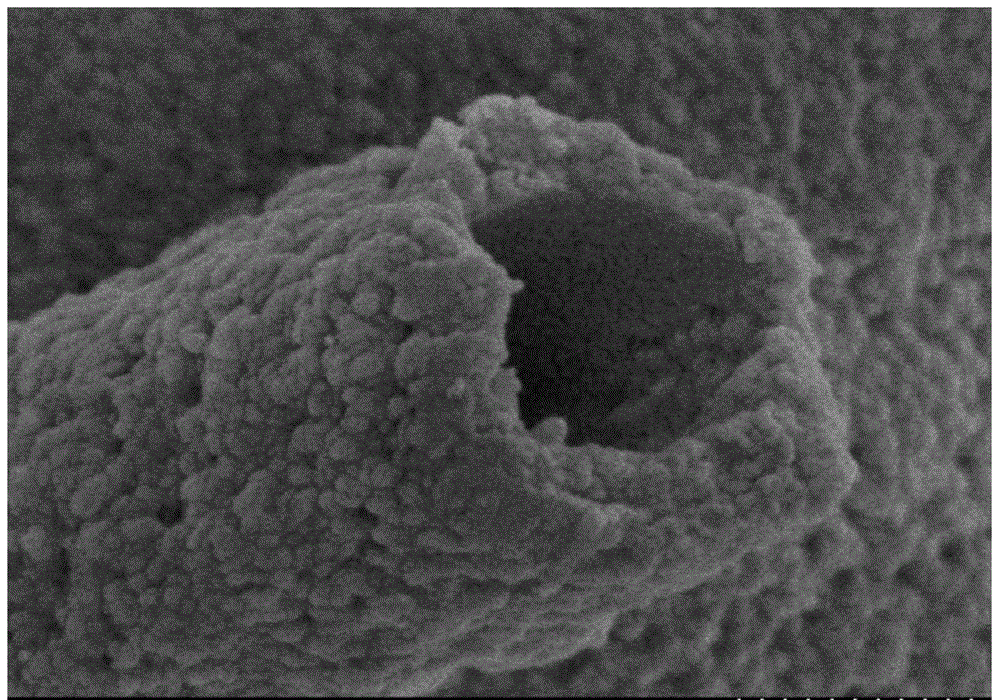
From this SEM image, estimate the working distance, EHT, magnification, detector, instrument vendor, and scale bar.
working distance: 9.3 mm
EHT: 3 kV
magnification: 60 K X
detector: SE(M)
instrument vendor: Hitachi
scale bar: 500 nm